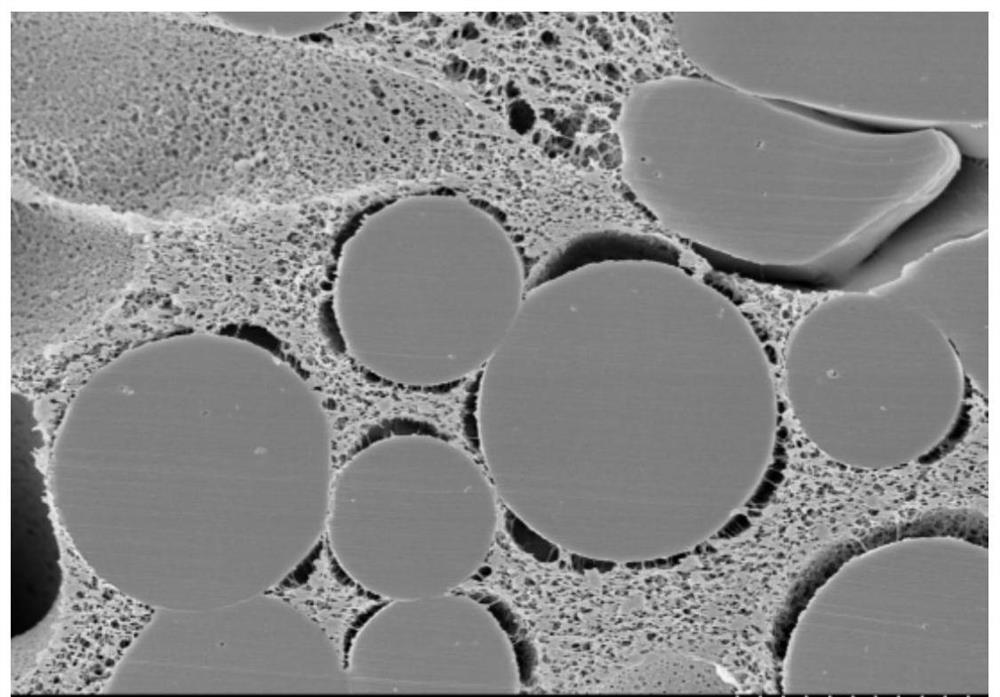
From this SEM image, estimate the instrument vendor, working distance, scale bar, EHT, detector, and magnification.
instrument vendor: Hitachi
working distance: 7.5 mm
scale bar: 10000 nm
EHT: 5 kV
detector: SE(M)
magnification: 3 K X